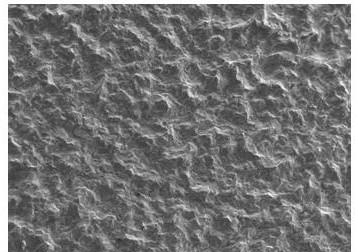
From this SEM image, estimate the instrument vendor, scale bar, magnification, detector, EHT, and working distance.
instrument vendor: JEOL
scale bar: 100000 nm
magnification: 0.07 K X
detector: SEI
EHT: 20 kV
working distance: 10 mm